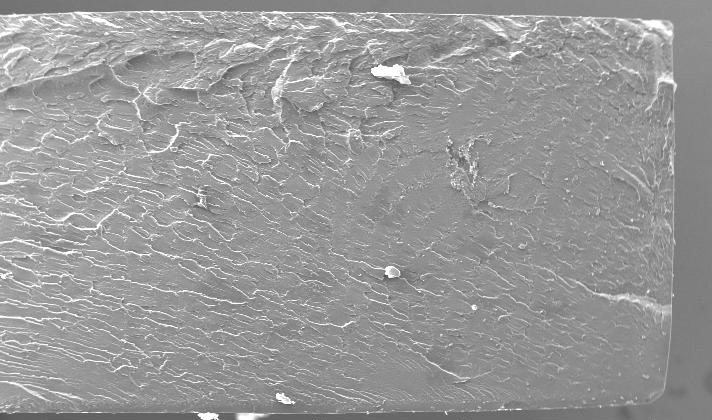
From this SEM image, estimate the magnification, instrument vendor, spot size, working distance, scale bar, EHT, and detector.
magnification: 0.05 K X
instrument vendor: FEI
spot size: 5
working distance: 10.6 mm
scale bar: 1000000 nm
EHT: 20 kV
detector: SE